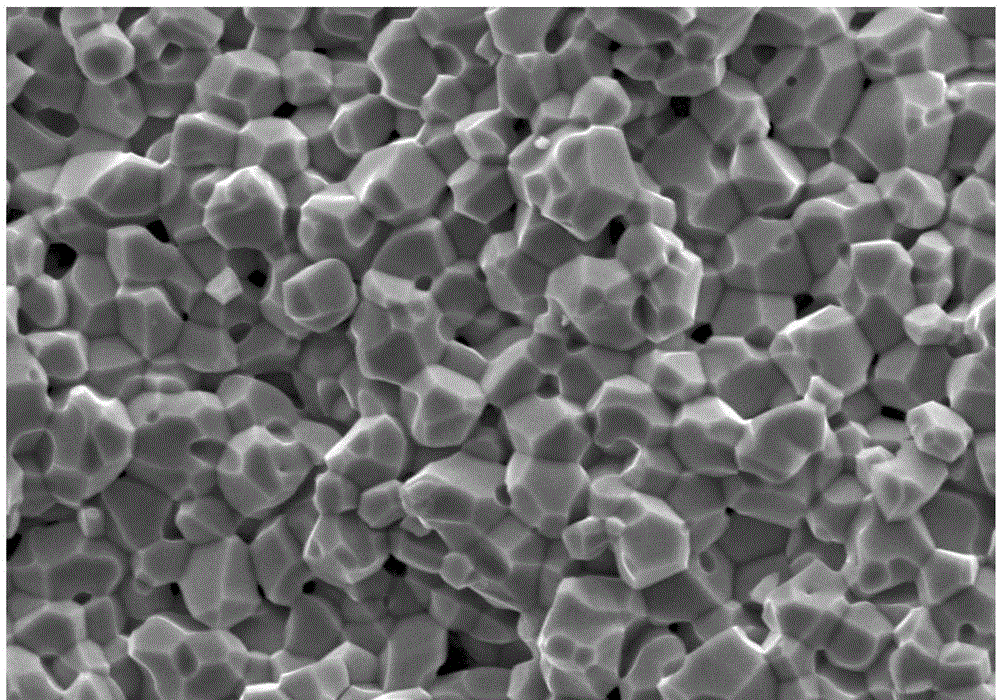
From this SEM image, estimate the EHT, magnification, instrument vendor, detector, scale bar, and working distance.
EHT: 15 kV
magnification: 2 K X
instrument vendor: JEOL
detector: SEI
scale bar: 10000 nm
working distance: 8 mm